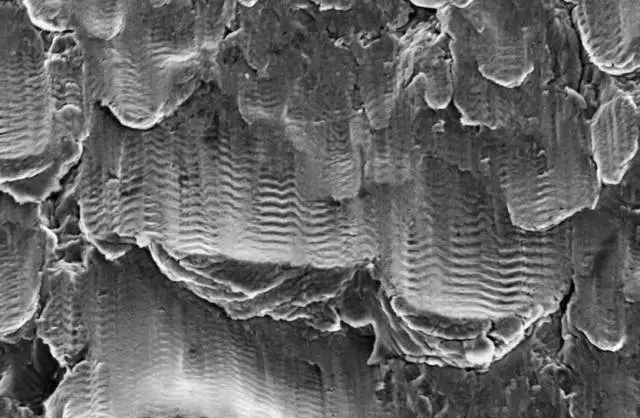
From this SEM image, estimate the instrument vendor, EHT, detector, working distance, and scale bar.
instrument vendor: Zeiss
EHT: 20 kV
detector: SE2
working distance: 29 mm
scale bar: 20000 nm